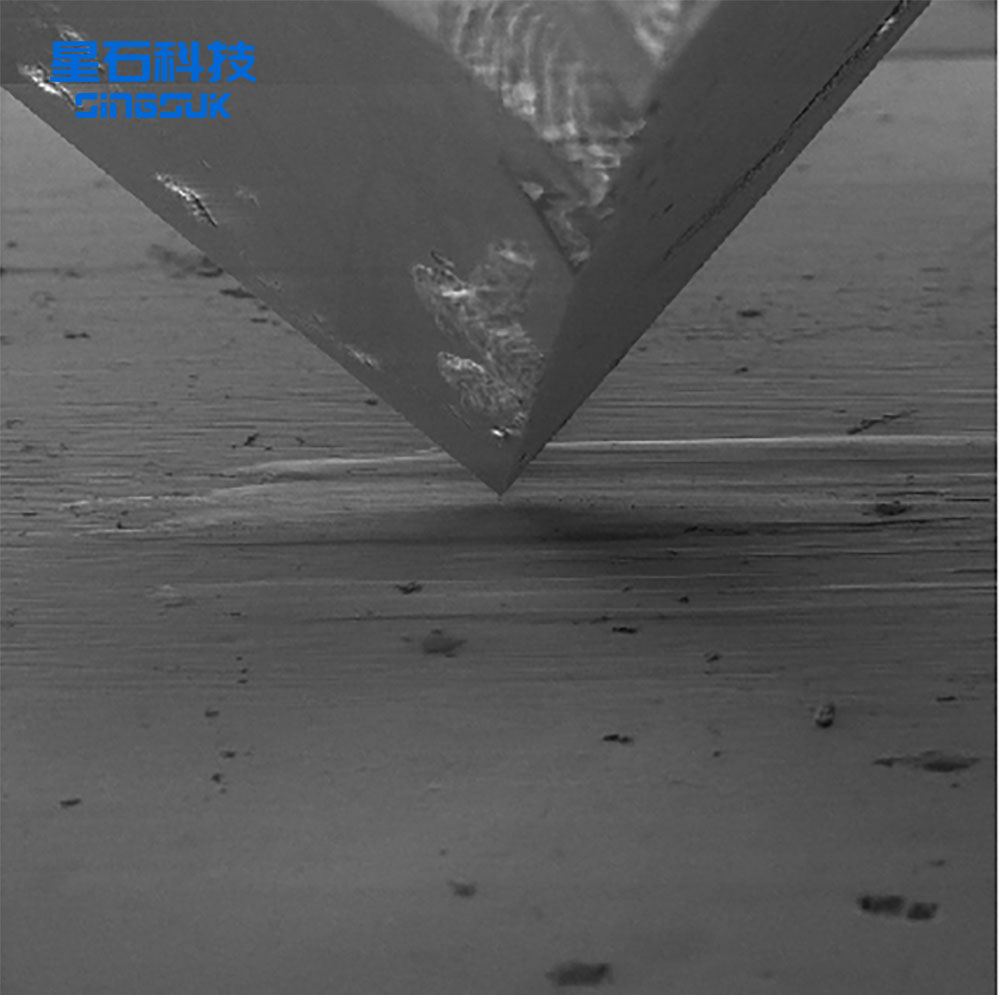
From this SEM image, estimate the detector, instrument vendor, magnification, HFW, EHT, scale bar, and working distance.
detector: SE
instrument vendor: Tescan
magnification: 1.33 K X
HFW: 217 µm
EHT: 5 kV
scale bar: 50000 nm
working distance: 26.35 mm